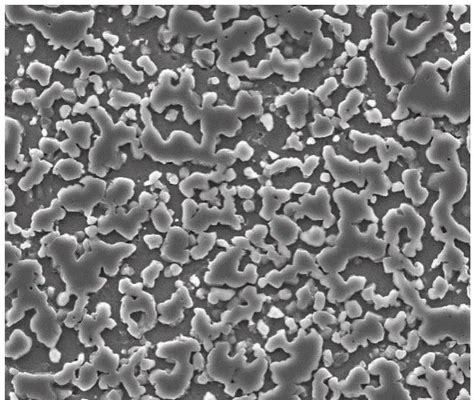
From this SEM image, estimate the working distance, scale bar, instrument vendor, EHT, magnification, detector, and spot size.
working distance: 10.4 mm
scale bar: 50000 nm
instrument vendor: FEI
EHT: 20 kV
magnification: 2 K X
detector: ETD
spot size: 4